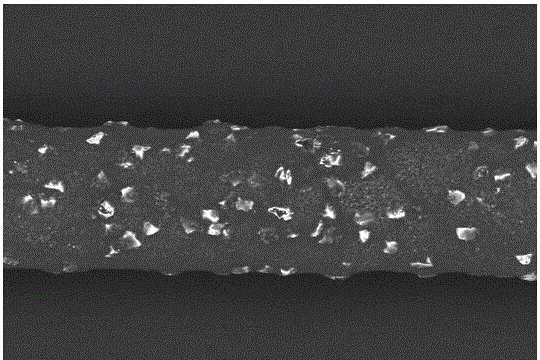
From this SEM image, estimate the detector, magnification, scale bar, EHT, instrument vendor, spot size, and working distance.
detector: BEC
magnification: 0.3 K X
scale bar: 50000 nm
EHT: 15 kV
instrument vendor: JEOL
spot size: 50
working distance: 10 mm